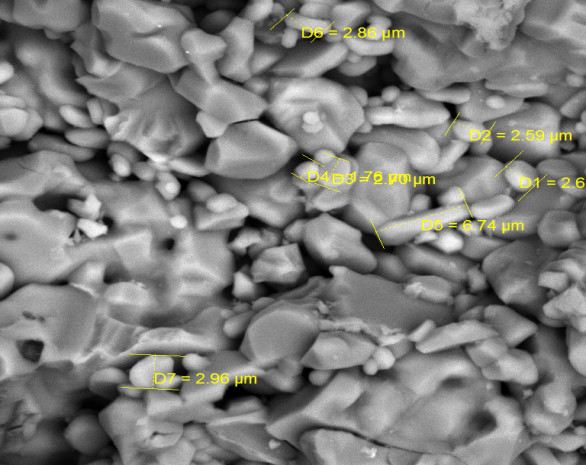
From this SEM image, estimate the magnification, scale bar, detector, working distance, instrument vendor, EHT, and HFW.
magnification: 5.07 K X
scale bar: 10000 nm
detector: BSE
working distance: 15.16 mm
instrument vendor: Tescan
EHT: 20 kV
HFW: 41 µm